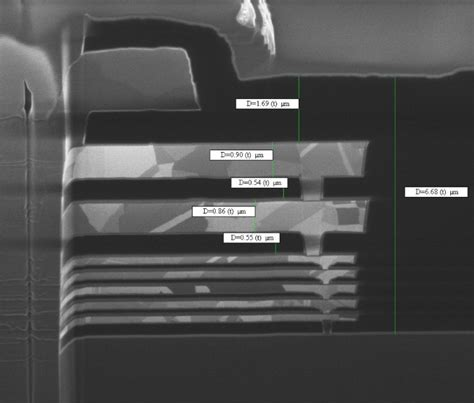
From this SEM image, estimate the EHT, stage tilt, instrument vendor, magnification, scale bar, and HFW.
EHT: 30 kV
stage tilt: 45°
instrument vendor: FEI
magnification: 35 K X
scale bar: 2000 nm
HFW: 8.69 µm